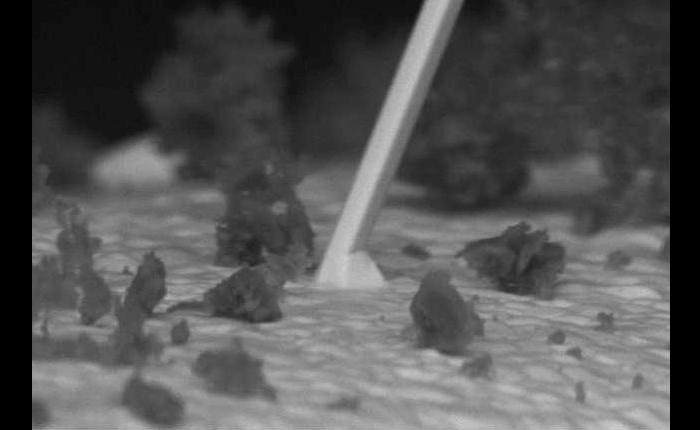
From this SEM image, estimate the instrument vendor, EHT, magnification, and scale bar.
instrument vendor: JEOL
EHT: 10 kV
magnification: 3.5 K X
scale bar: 5000 nm